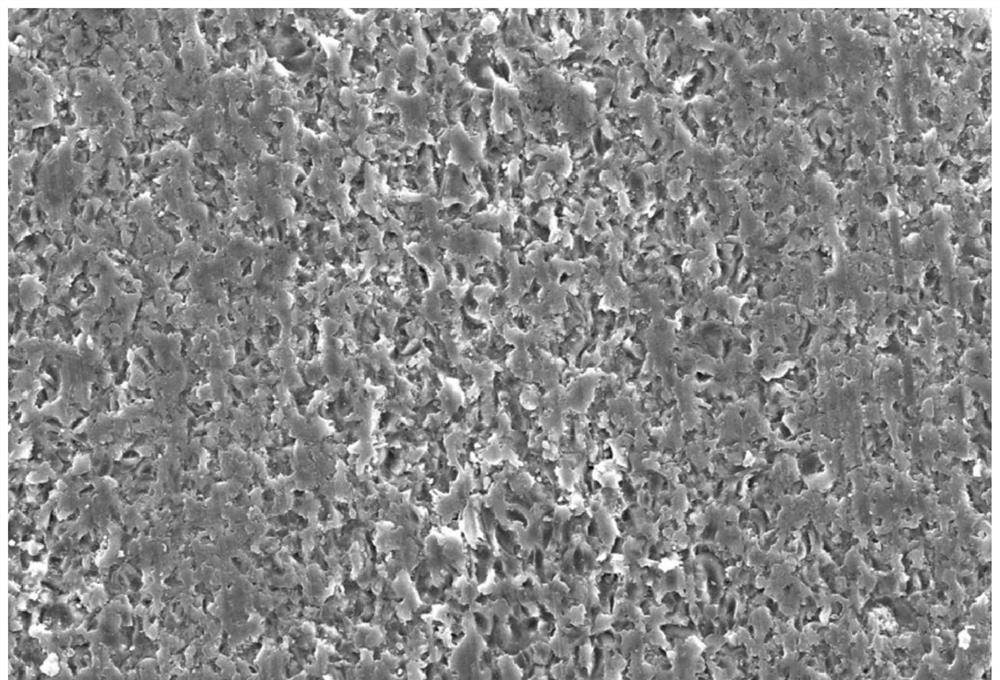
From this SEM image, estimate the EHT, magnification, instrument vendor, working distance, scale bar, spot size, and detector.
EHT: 20 kV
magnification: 0.5 K X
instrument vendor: JEOL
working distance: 9 mm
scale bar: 50000 nm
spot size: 50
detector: SEI